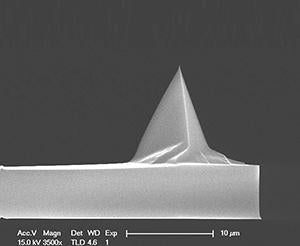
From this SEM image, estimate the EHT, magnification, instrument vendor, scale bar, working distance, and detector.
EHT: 15 kV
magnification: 3.5 K X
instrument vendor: FEI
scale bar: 10000 nm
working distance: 4.6 mm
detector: TLD